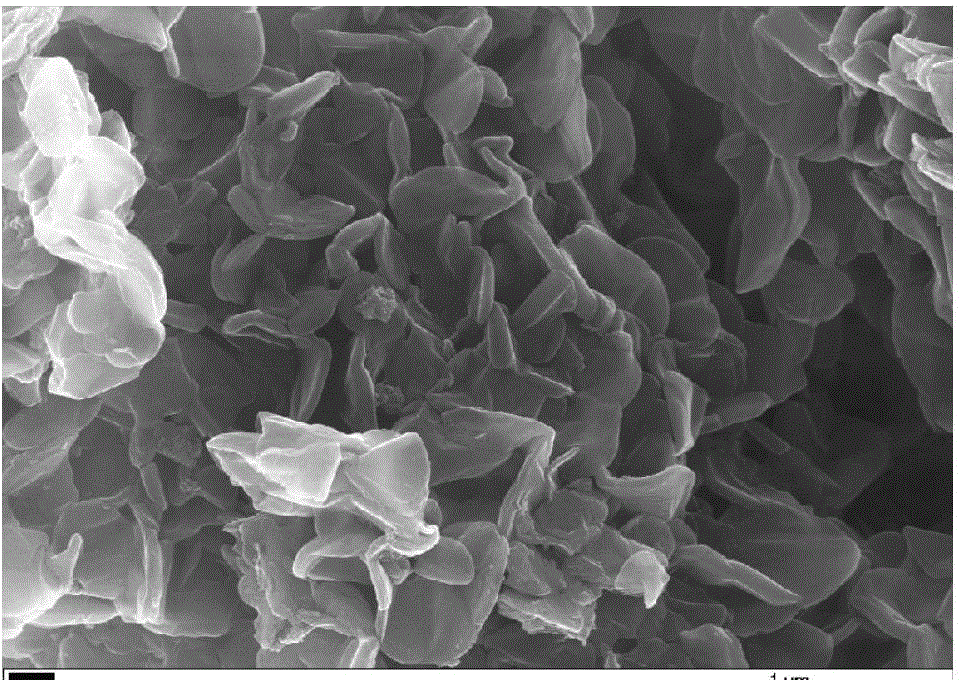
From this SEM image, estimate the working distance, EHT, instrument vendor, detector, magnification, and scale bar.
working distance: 5.8 mm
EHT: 20 kV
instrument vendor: Zeiss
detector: InLens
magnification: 50 K X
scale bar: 1000 nm